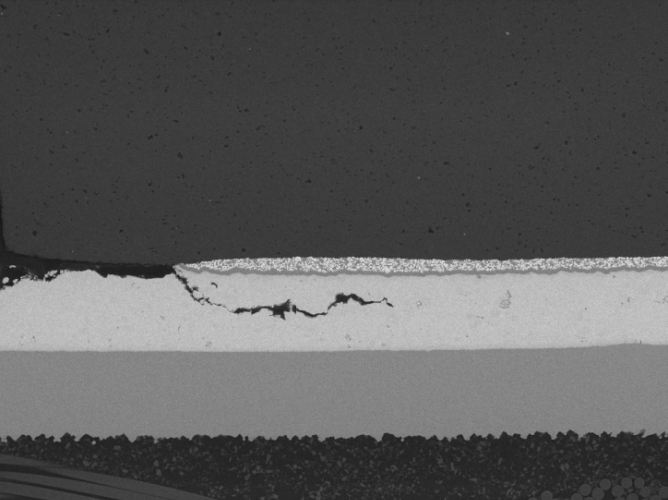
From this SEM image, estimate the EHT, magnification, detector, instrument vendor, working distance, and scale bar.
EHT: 20 kV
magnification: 0.502 K X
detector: BSE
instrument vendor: Tescan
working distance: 14.72 mm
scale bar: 100000 nm